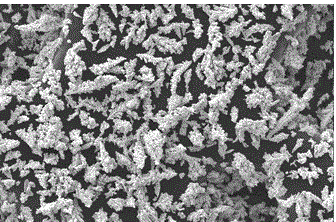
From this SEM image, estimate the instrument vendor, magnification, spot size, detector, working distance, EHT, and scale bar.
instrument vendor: FEI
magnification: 0.5 K X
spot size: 4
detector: SE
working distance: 9.9 mm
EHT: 22 kV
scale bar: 100000 nm